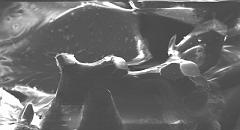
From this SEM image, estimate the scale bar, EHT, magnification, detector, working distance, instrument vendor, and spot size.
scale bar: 100000 nm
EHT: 20 kV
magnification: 0.625 K X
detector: SE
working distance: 10.4 mm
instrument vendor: FEI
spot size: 3